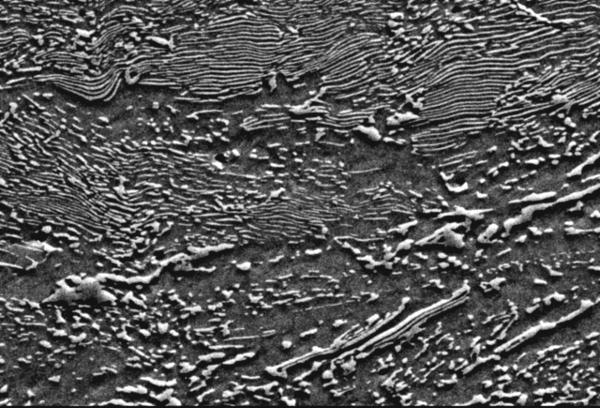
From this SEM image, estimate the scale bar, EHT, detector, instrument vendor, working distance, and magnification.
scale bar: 20000 nm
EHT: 20 kV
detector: SE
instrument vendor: JEOL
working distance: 19.9 mm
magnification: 2.5 K X